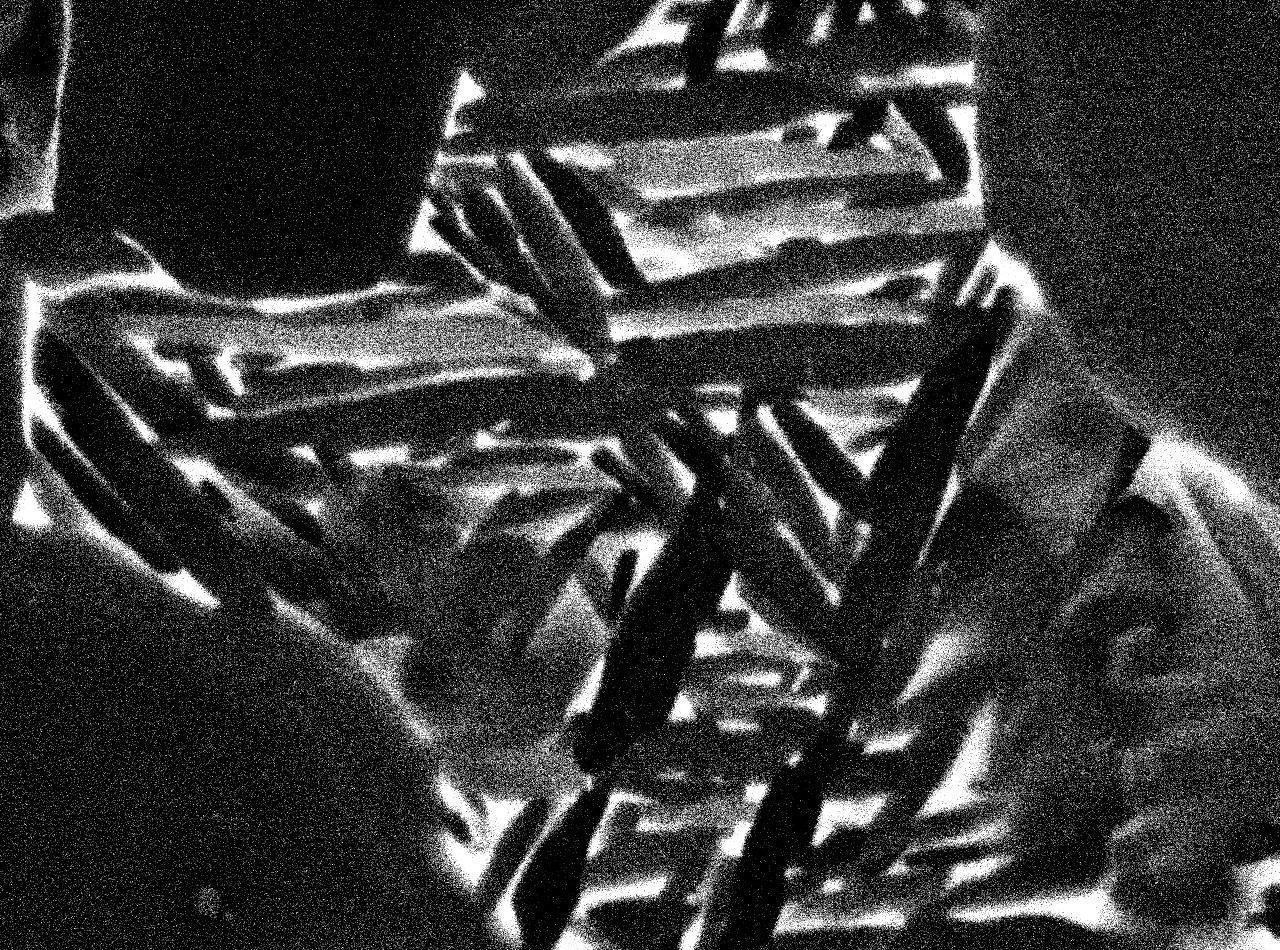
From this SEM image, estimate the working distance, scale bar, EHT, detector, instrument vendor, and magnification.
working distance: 9.9 mm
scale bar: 1000 nm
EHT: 15 kV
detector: COMPO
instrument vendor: JEOL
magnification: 10 K X